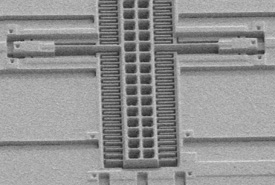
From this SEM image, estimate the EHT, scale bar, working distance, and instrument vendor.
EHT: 1 kV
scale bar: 50000 nm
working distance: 12.9 mm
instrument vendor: Hitachi